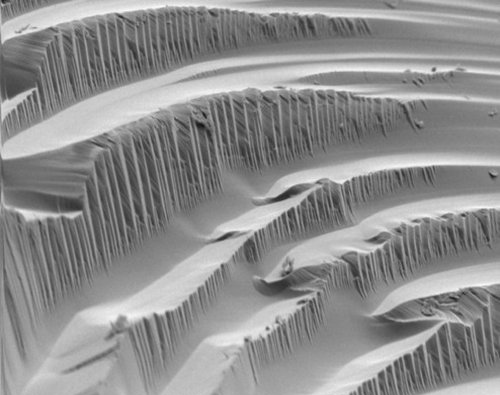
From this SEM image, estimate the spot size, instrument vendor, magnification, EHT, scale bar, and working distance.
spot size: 3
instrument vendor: FEI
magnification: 1.61 K X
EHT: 5 kV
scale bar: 20000 nm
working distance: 5.4 mm